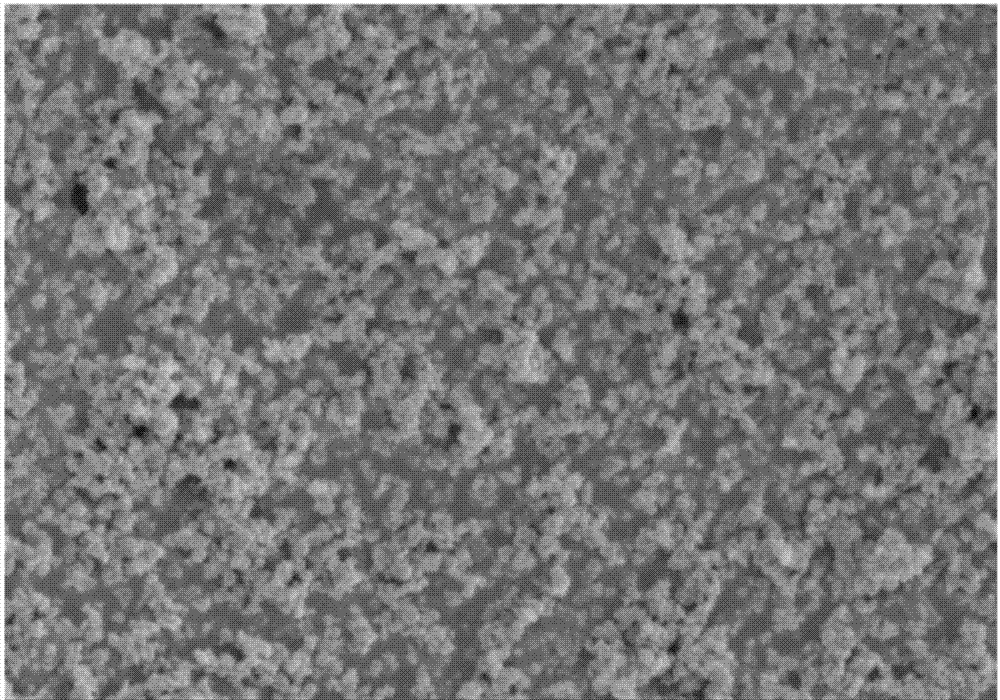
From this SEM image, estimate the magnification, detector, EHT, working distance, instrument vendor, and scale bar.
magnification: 20 K X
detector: SE(M)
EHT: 3 kV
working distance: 8.5 mm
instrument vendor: Hitachi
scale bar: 2000 nm